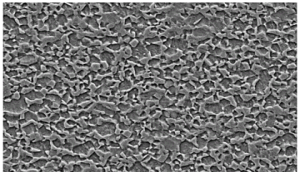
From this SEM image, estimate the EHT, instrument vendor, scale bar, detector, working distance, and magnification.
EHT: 15 kV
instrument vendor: Zeiss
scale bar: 2000 nm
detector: SE2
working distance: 10 mm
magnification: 2 K X